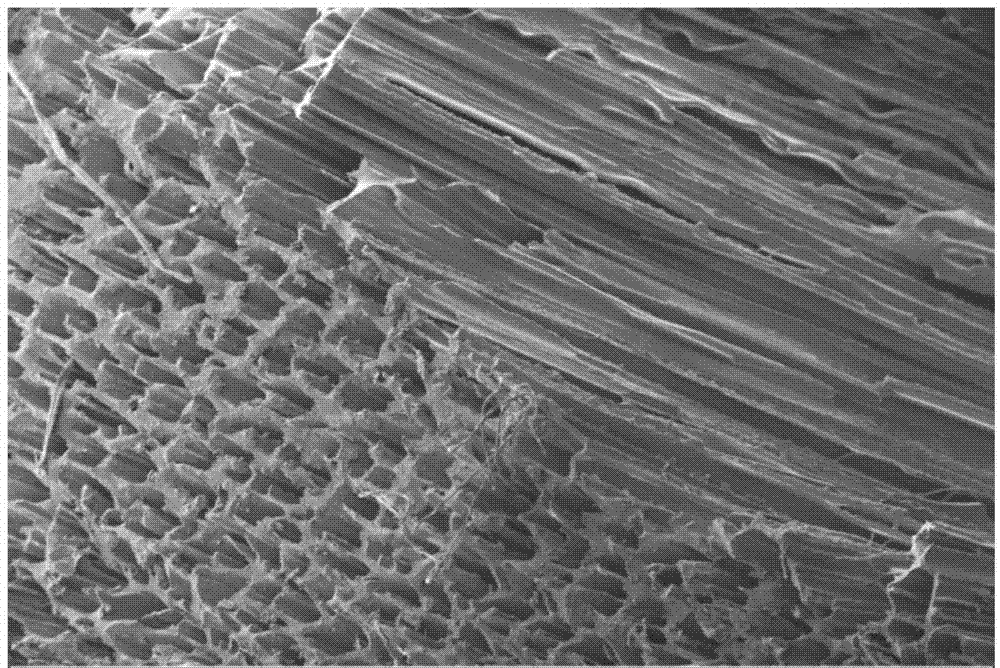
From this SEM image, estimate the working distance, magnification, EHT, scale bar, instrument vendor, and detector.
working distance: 16 mm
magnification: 0.025 K X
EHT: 10 kV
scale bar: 200000 nm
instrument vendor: Zeiss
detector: SE2